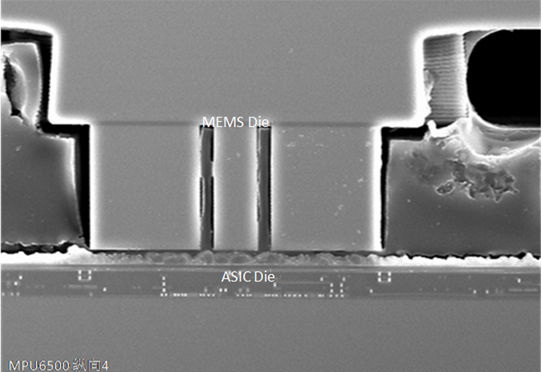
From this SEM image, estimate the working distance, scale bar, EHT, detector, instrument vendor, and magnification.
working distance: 11.6 mm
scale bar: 50000 nm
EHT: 20 kV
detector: SE(M)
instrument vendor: Hitachi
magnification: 1 K X